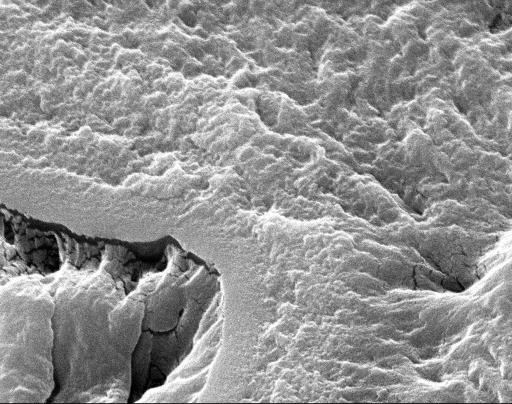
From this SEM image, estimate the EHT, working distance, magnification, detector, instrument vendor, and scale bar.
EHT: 18 kV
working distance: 6.2 mm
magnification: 10.378 K X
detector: SE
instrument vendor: Zeiss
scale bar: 3000 nm